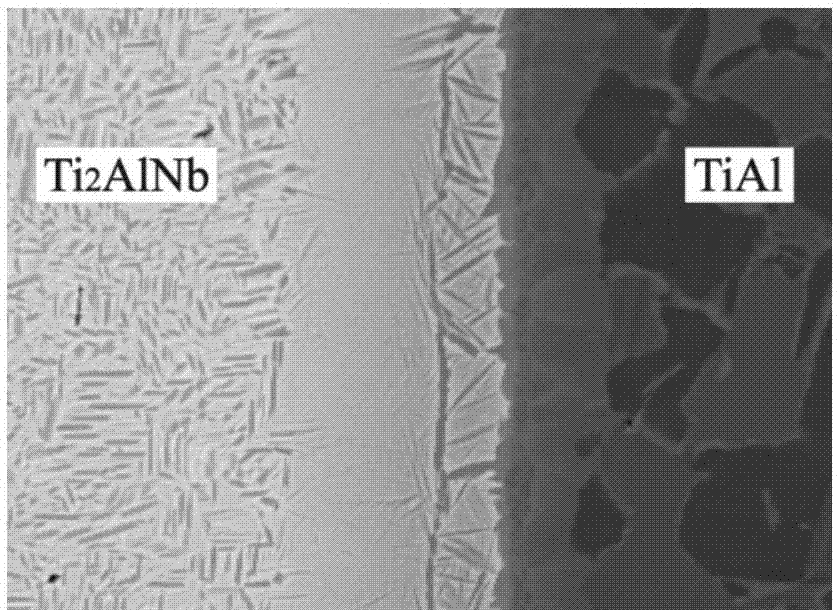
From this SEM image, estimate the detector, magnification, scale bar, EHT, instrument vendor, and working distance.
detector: COMP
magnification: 1.6 K X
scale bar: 10000 nm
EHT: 20 kV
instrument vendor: JEOL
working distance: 11 mm